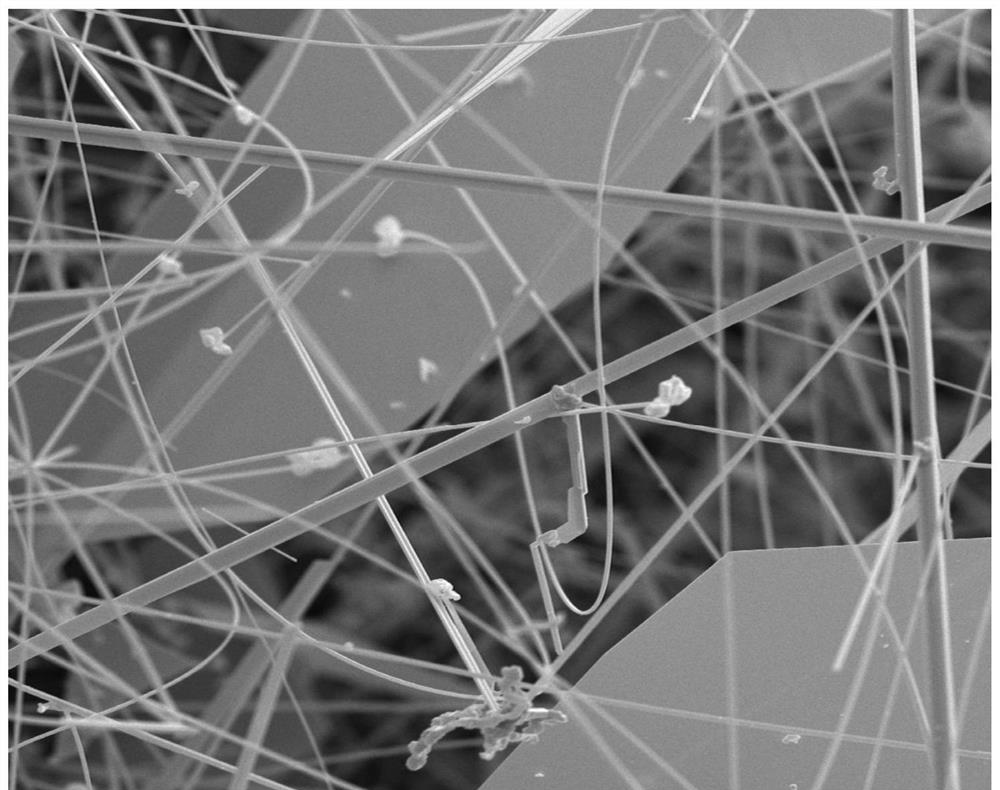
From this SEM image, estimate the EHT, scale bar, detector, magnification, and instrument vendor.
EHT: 10 kV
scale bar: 20000 nm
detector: SED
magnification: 3.8 K X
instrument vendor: other